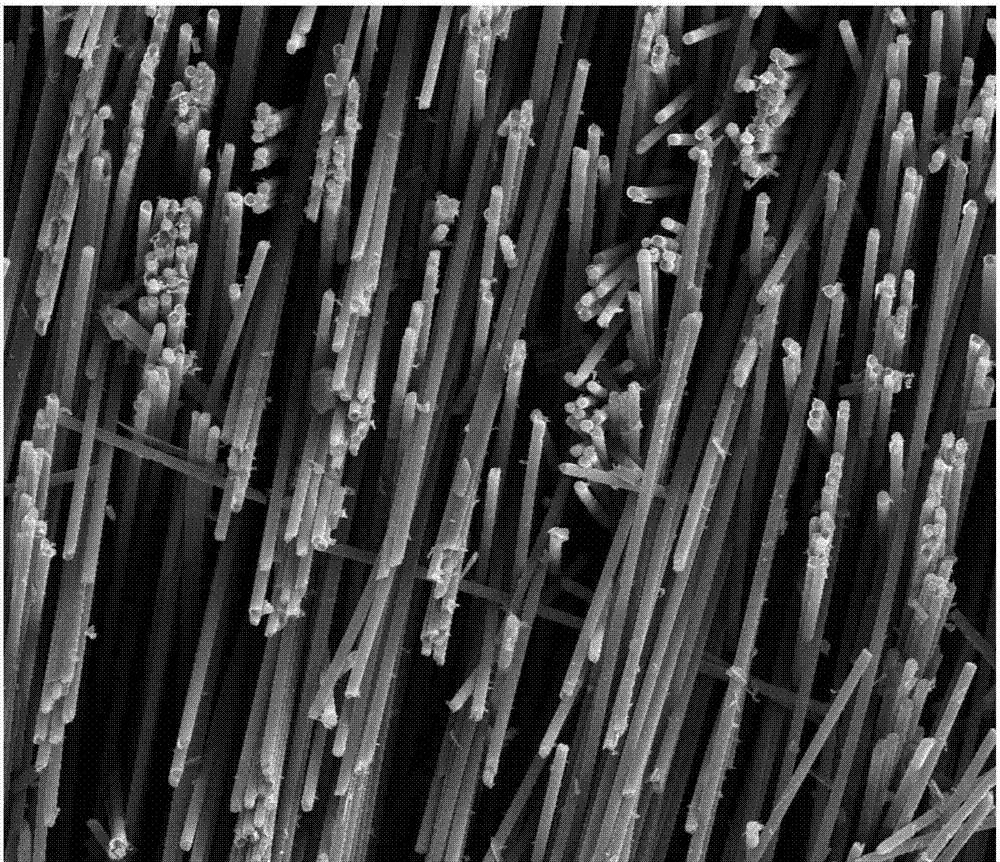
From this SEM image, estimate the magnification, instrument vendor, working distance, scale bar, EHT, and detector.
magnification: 0.5 K X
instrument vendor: FEI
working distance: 5.8 mm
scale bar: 100000 nm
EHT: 30 kV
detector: SE